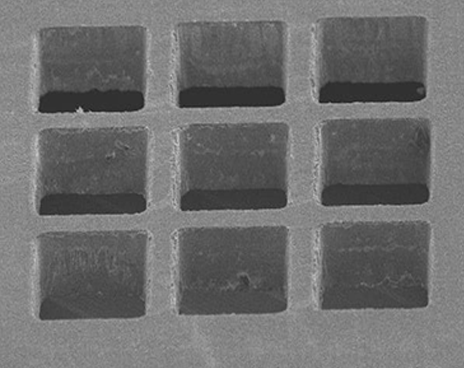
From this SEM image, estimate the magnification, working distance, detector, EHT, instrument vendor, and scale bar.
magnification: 0.18 K X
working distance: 36.7 mm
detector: SE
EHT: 10 kV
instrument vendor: Hitachi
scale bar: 300000 nm